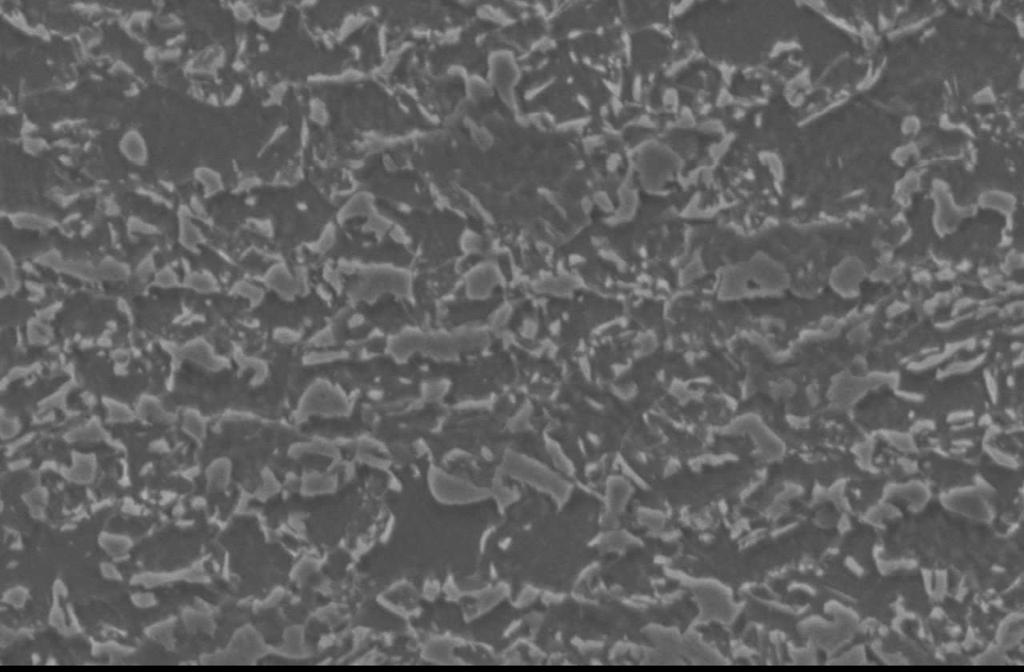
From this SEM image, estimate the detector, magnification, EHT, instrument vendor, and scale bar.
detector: SEI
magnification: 2.5 K X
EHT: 15 kV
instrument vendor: JEOL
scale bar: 10000 nm